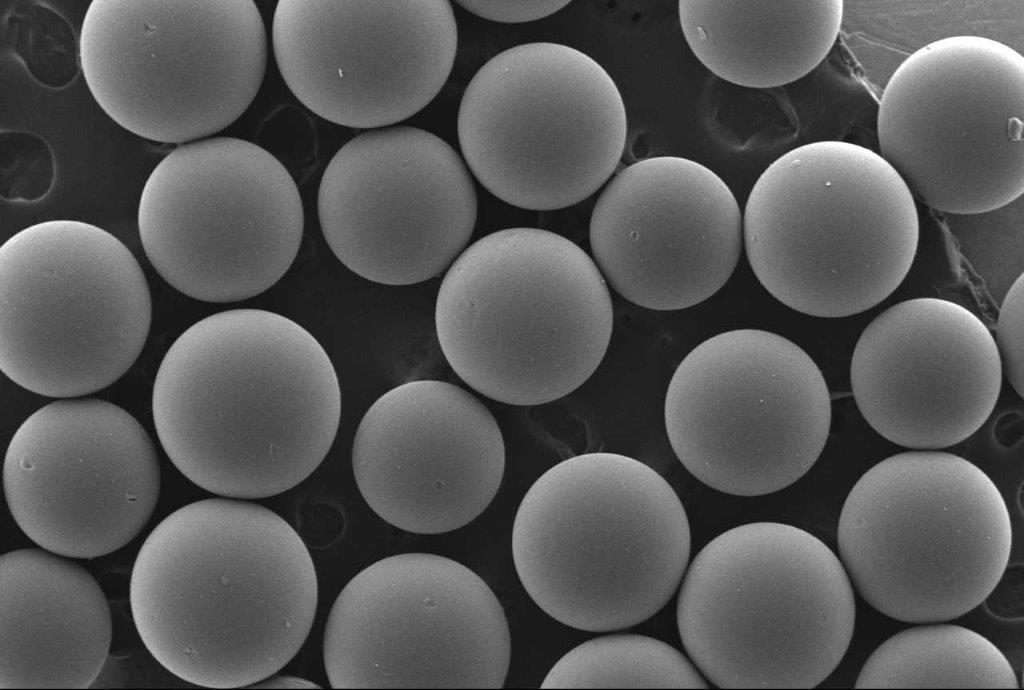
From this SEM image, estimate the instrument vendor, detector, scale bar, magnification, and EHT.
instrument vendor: JEOL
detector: SEI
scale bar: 500000 nm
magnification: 0.04 K X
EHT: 20 kV